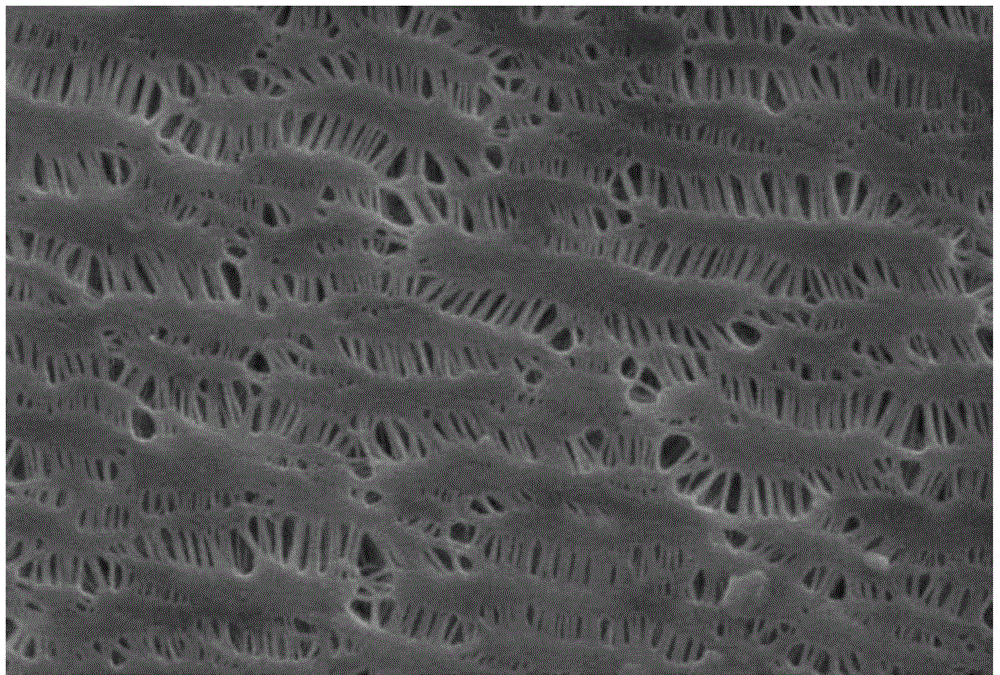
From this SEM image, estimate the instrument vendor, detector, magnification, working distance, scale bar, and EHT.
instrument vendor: Zeiss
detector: SE1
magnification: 20 K X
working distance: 6 mm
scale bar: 200 nm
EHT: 15 kV